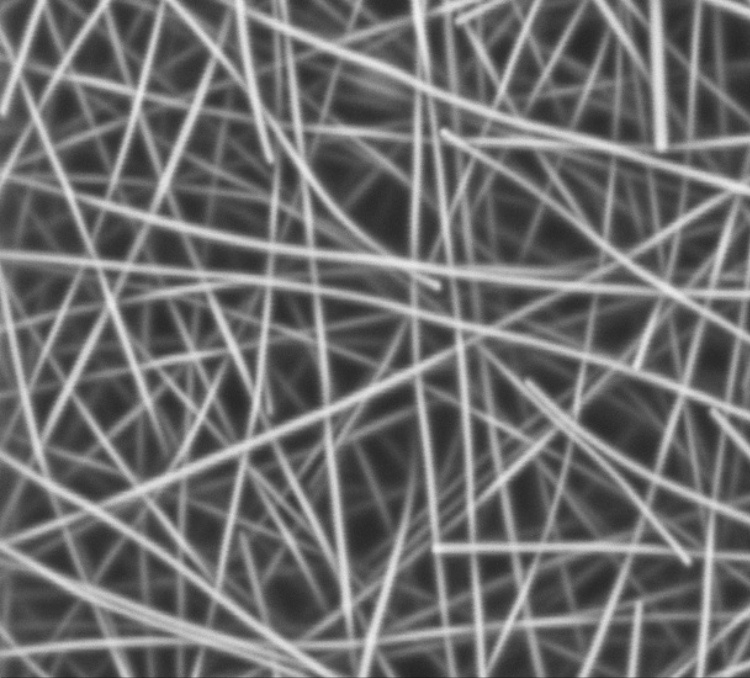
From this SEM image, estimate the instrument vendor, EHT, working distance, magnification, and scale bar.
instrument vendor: Zeiss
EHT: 3 kV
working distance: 5.1 mm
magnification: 30 K X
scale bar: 200 nm